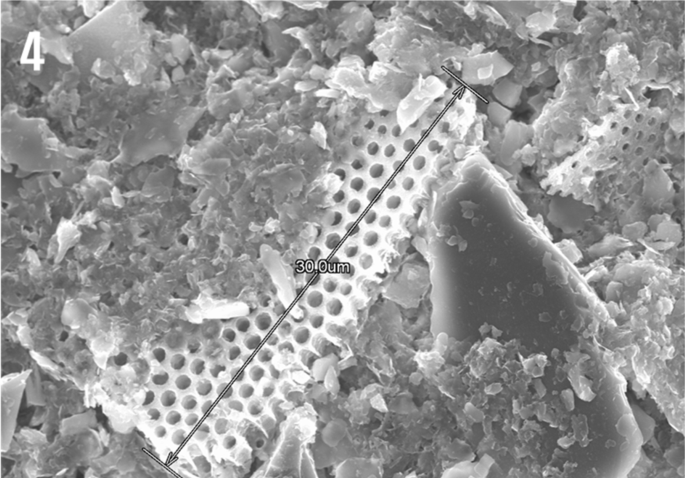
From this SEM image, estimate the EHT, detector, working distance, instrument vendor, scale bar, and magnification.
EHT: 15 kV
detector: SE(U)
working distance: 11.8 mm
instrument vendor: Hitachi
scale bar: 10000 nm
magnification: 3 K X